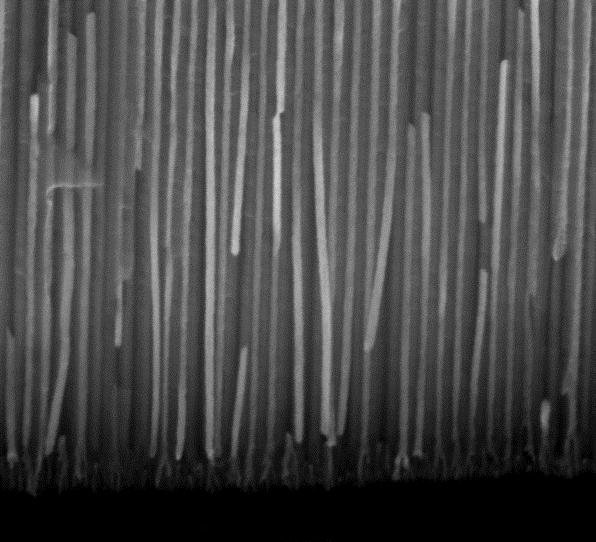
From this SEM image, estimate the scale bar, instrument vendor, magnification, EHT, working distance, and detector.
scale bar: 2000 nm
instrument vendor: FEI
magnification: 70 K X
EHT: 15 kV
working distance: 9.9 mm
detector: ETD